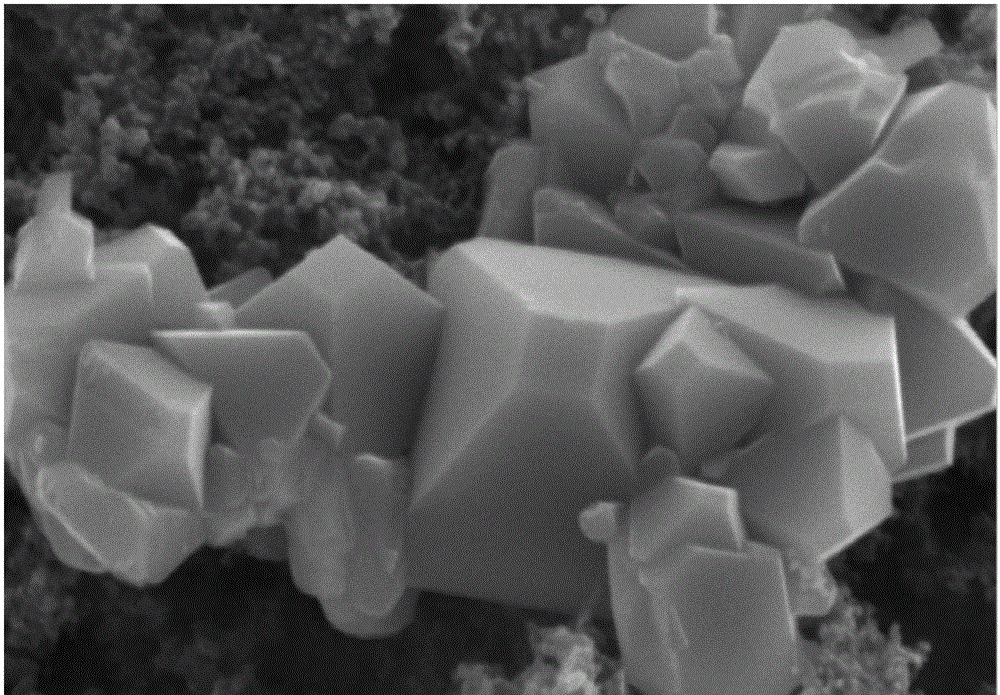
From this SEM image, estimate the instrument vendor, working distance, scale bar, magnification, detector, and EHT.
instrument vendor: Hitachi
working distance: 10 mm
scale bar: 3000 nm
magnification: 15 K X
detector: SE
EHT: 15 kV